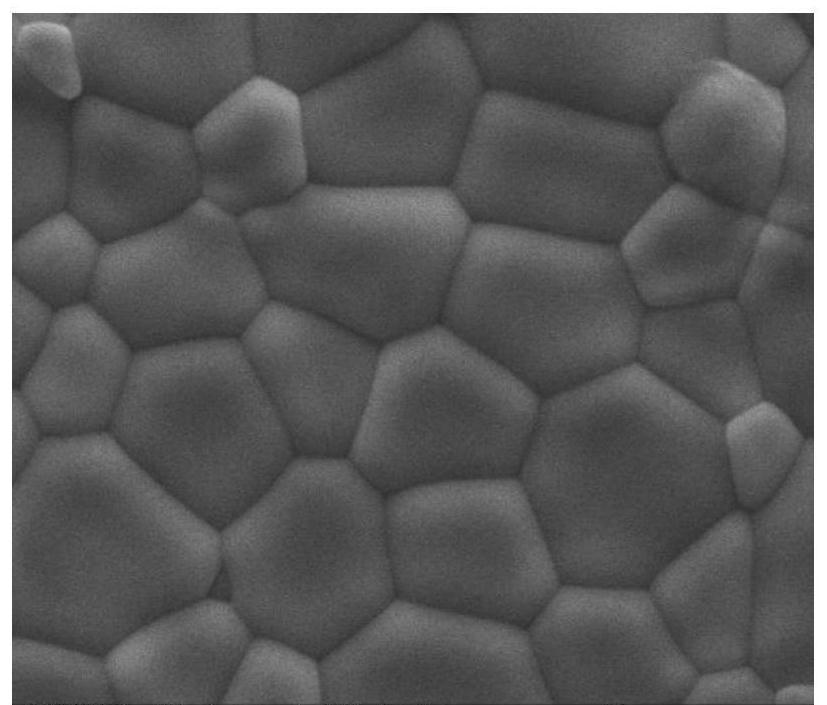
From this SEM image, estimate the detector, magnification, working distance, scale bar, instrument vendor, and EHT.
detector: SE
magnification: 10 K X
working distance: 9.2 mm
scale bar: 10000 nm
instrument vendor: FEI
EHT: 20 kV